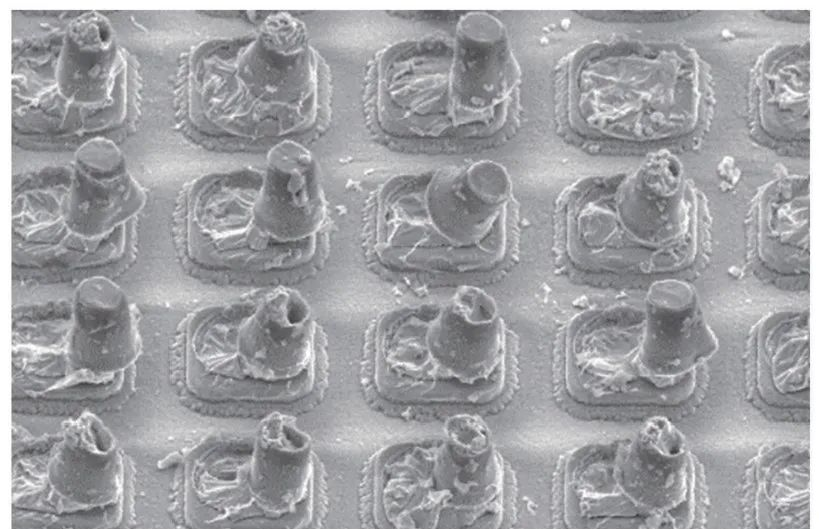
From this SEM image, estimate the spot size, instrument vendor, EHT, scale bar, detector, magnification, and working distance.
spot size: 3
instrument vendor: FEI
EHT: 5 kV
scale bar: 5000 nm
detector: SE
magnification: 4 K X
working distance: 5.2 mm